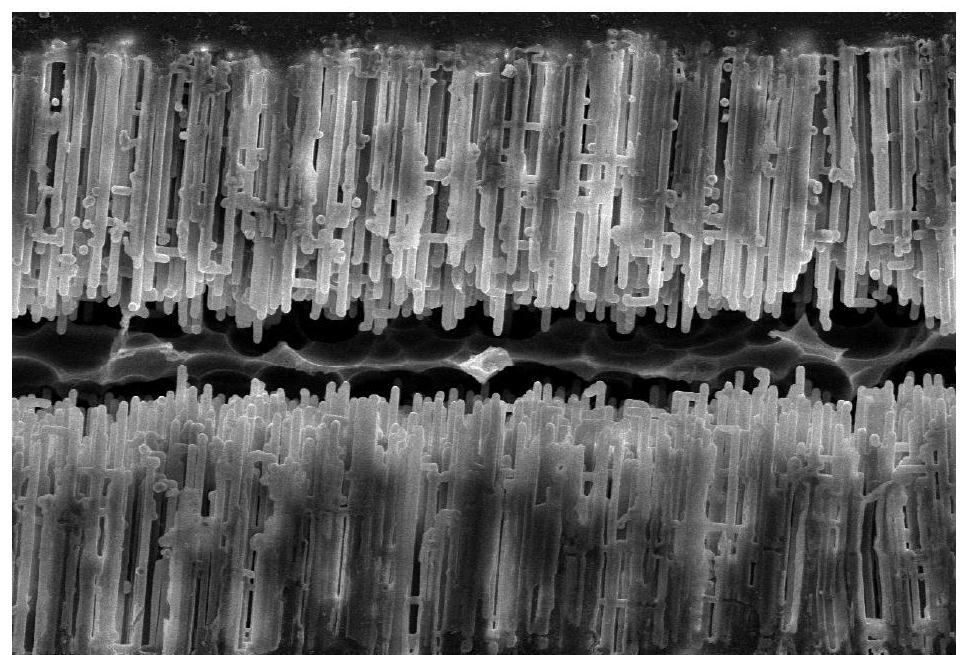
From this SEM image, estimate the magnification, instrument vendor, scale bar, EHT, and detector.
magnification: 0.7 K X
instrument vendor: JEOL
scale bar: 20000 nm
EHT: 20 kV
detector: SEI